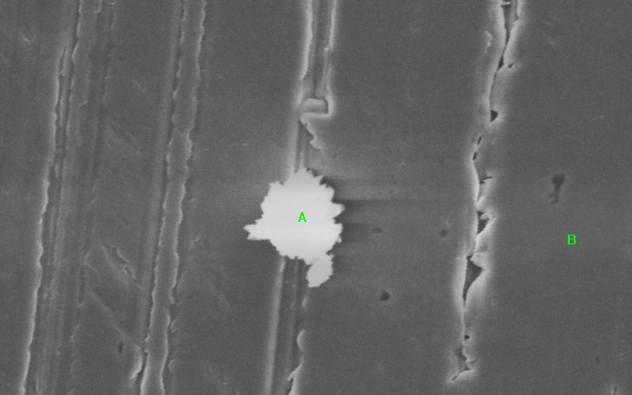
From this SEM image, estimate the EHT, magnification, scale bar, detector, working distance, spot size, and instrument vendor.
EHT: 20 kV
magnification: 2 K X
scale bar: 5000 nm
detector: SE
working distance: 43 mm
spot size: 4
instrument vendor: FEI